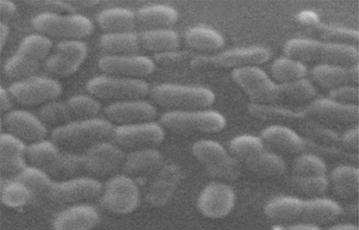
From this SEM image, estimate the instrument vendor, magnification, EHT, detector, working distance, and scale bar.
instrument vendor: Zeiss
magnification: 12 K X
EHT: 15 kV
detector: SE1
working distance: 25 mm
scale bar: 300 nm